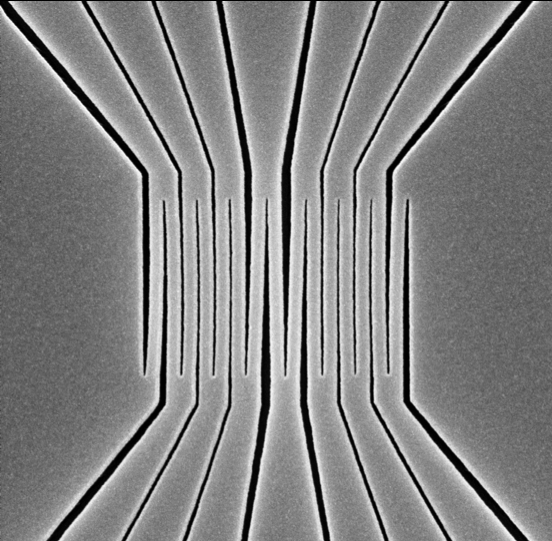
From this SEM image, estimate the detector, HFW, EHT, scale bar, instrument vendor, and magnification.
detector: SED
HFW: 5.37 µm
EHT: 10 kV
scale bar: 1000 nm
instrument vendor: other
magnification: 50 K X